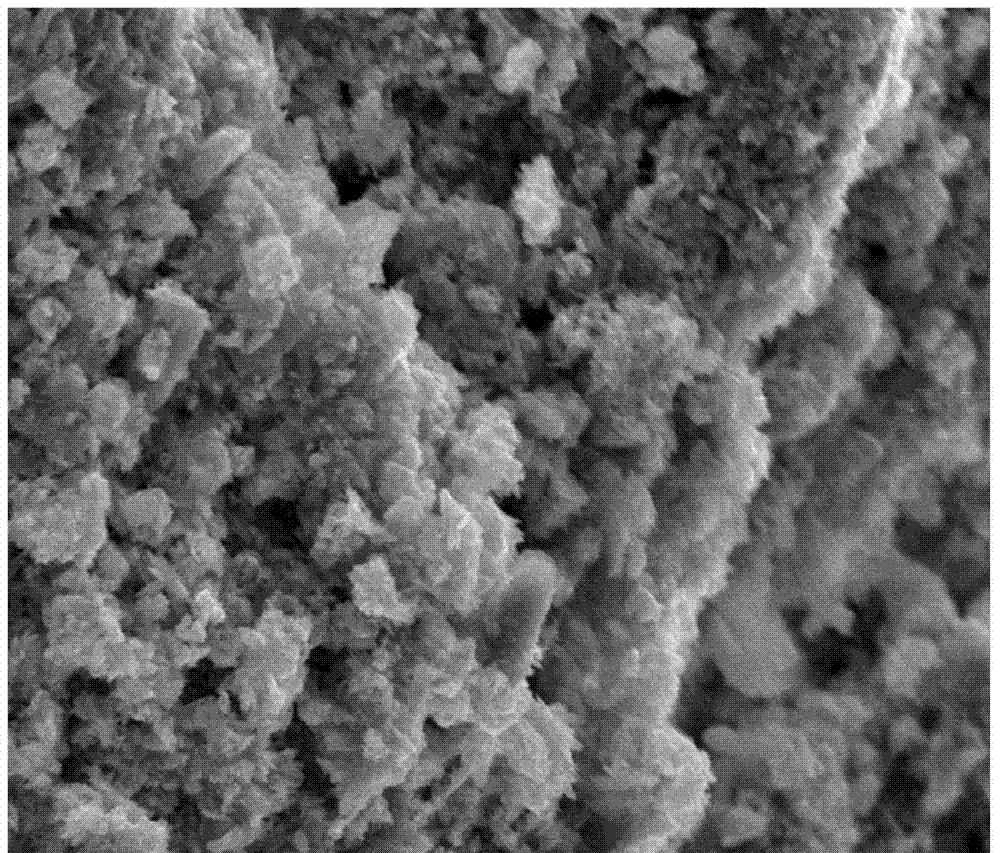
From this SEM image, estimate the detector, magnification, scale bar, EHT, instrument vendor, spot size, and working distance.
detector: ETD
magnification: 2.5 K X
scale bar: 20000 nm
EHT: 20 kV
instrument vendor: FEI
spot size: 3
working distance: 11.2 mm